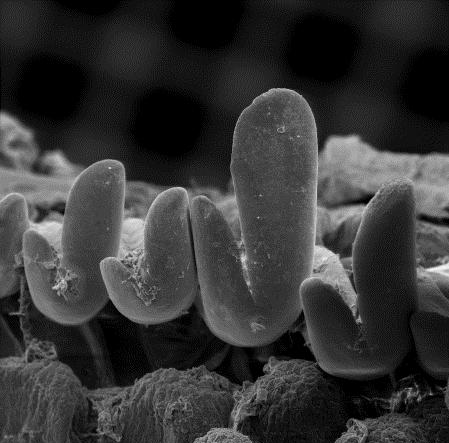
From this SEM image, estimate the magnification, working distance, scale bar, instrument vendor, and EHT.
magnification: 0.092 K X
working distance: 10 mm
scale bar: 200000 nm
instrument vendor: other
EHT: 15 kV